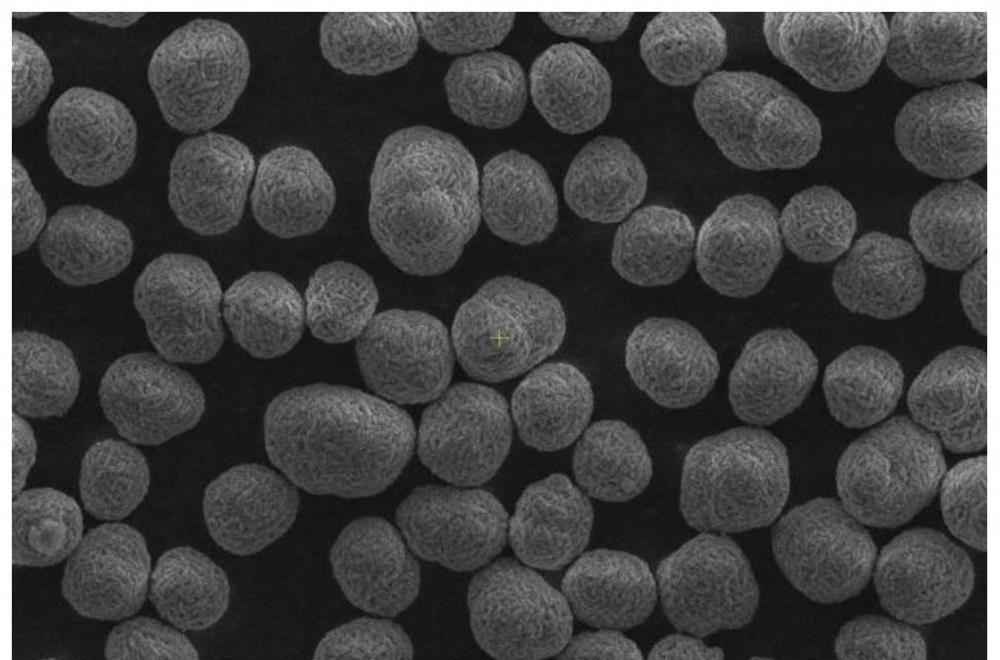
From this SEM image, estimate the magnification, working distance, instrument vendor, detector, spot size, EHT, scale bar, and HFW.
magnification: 5 K X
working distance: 11.6 mm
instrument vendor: Thermo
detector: ETD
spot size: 6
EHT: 6 kV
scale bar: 10000 nm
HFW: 41.4 µm